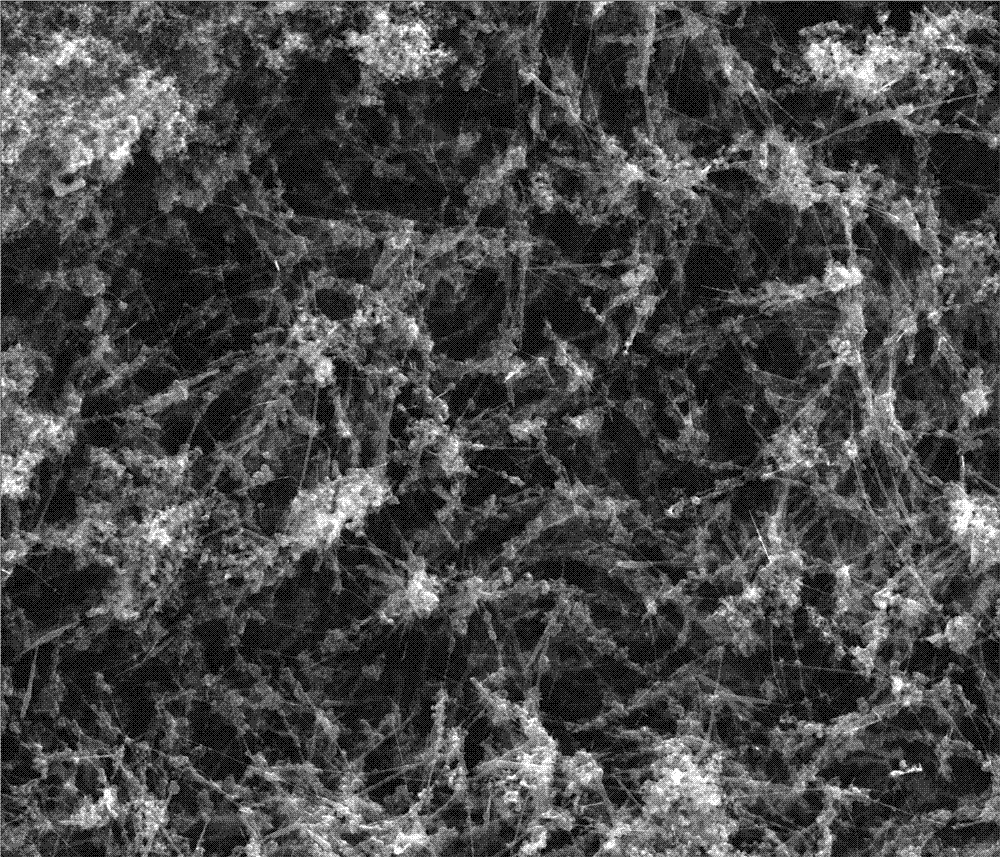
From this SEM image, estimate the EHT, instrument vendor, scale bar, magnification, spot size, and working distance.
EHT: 15 kV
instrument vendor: FEI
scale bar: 5000 nm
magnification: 16.858 K X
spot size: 3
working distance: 9.8 mm